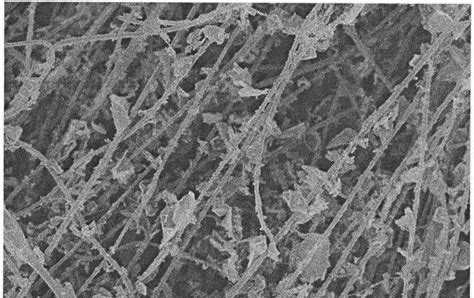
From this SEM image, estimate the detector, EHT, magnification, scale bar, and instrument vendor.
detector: SE(M)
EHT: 10 kV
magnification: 5 K X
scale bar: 10000 nm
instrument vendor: Hitachi